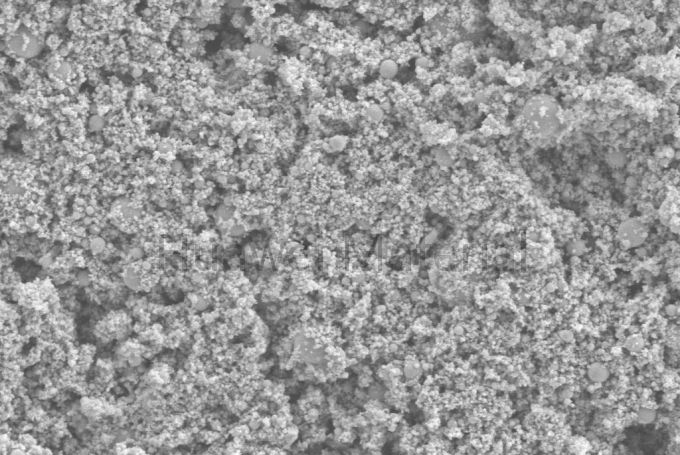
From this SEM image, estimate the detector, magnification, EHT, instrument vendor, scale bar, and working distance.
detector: SE2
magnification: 10 K X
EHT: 5 kV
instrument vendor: Zeiss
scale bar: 1000 nm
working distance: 8.6 mm